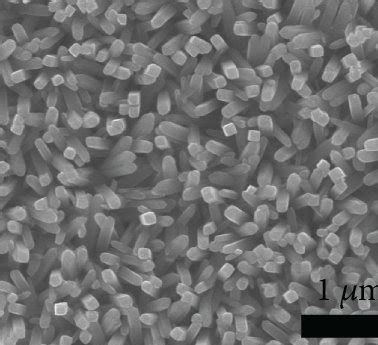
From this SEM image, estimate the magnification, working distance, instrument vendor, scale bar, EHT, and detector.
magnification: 25 K X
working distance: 10 mm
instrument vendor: JEOL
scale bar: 1000 nm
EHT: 15 kV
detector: LED